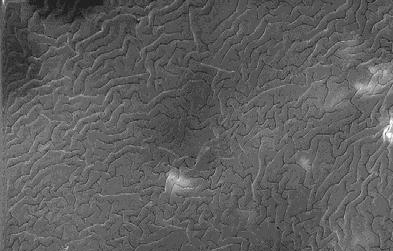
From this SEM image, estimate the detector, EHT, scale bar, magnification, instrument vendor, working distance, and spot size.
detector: SE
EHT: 25 kV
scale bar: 10000 nm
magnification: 2 K X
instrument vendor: FEI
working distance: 17.3 mm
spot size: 4